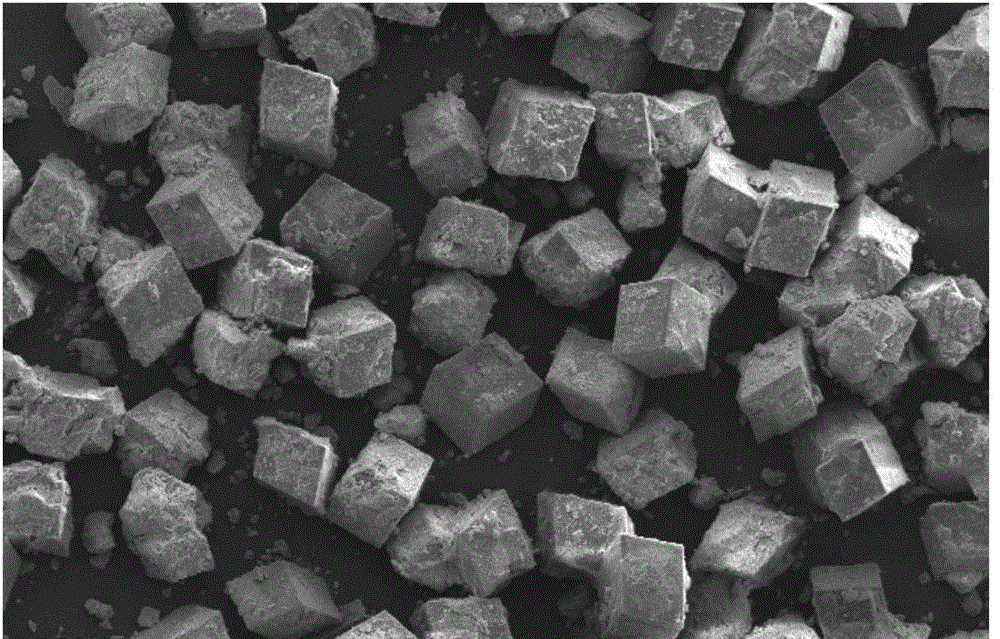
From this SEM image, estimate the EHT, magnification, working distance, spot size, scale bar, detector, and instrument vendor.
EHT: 10 kV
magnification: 0.2 K X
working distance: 5.8 mm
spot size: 3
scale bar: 100000 nm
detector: SE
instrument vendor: FEI